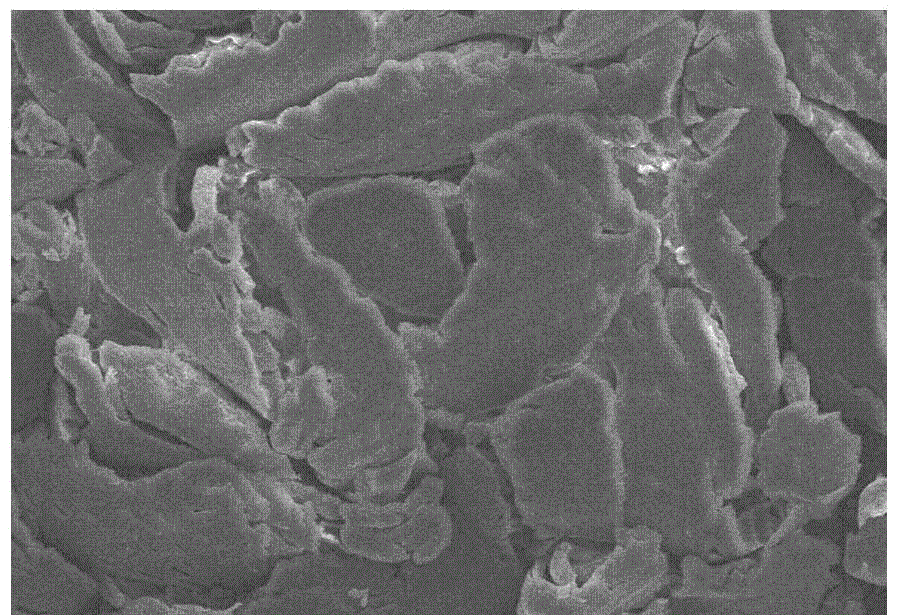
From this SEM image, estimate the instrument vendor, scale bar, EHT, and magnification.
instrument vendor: JEOL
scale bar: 50000 nm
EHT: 15 kV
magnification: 0.5 K X